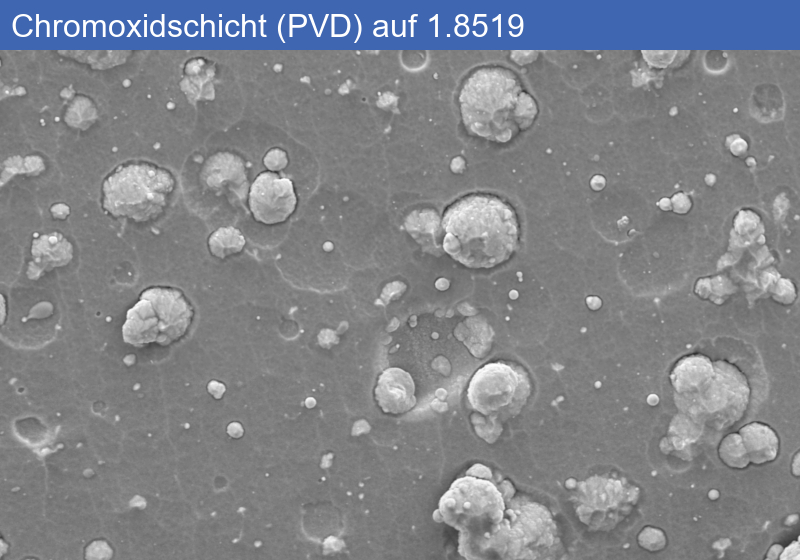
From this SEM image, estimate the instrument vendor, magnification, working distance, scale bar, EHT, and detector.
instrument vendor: Zeiss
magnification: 5 K X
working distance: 11.4 mm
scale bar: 1000 nm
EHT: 5 kV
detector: SE2